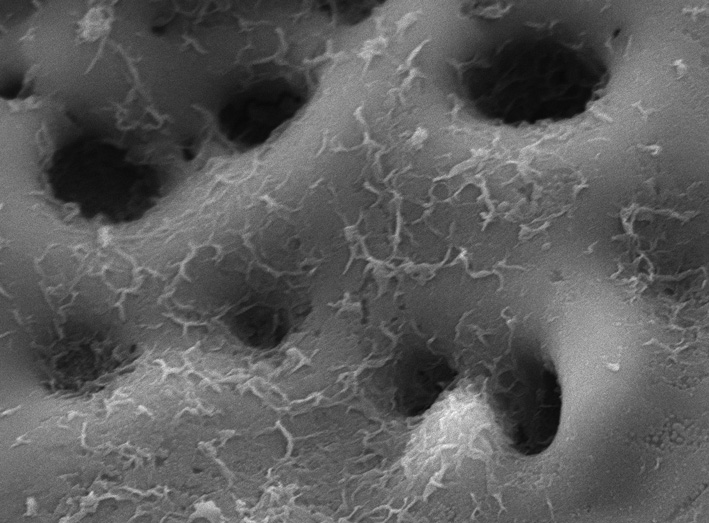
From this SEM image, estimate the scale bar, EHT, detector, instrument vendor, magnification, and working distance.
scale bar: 1000 nm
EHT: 10 kV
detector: LED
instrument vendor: JEOL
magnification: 20 K X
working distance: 6.7 mm